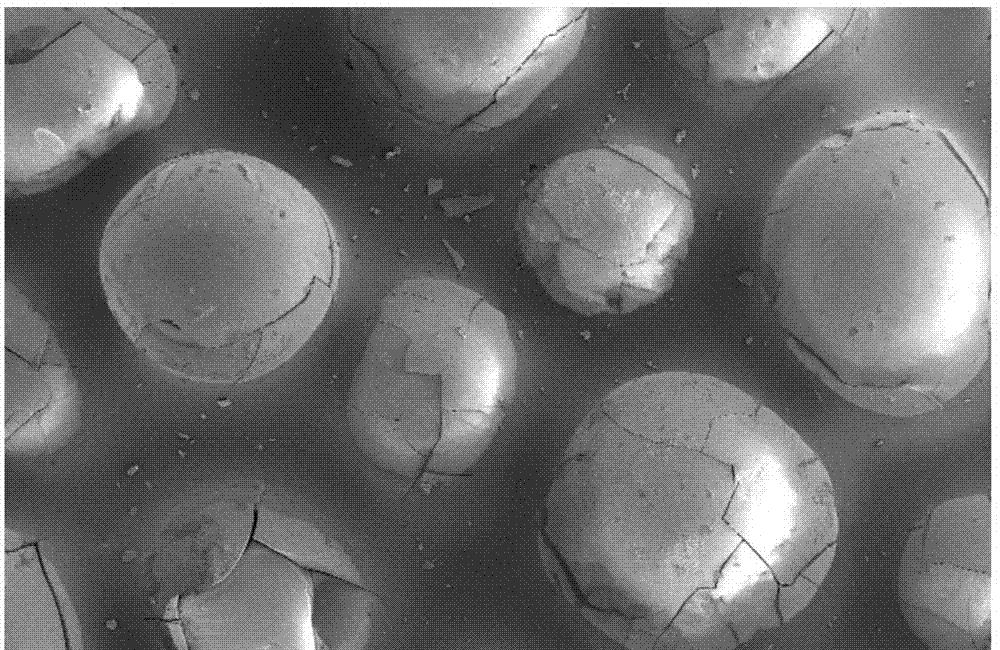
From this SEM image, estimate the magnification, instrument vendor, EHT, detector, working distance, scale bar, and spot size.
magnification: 0.1 K X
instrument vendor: FEI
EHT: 5 kV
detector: SE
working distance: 5.2 mm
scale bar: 500000 nm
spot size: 4